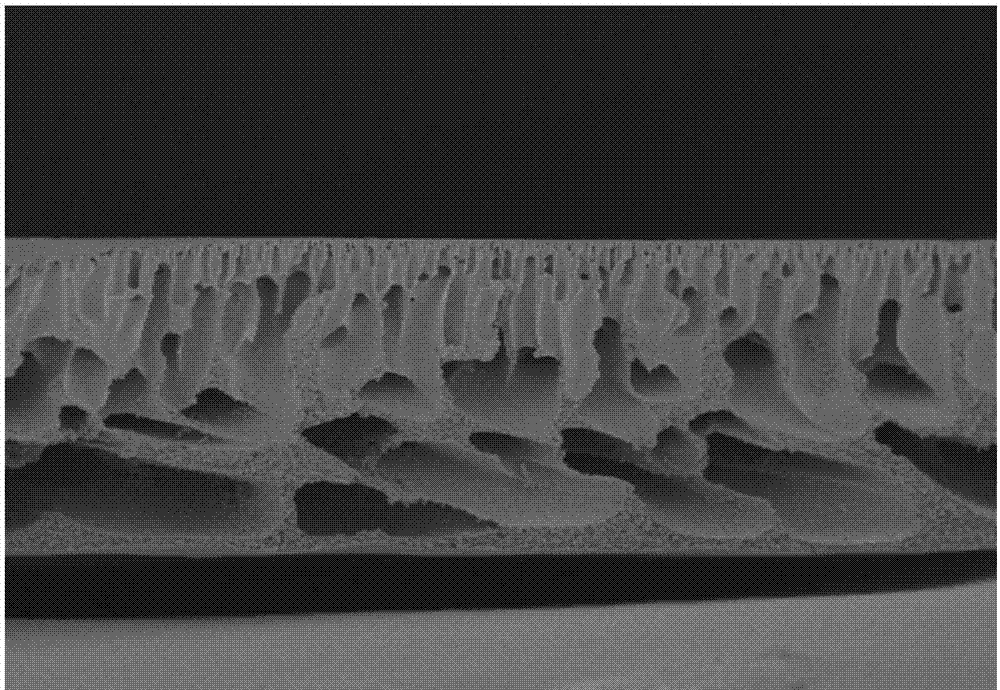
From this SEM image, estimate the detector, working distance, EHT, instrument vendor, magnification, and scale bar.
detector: SE(U)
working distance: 9 mm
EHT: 3 kV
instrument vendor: Hitachi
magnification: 1 K X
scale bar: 50000 nm